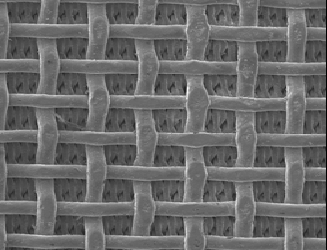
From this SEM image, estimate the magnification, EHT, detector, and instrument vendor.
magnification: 0.05 K X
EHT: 5 kV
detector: LEI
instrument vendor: JEOL